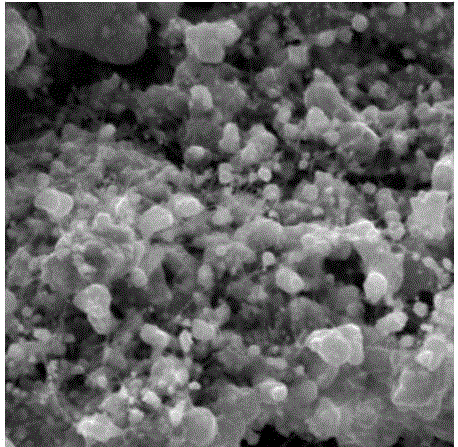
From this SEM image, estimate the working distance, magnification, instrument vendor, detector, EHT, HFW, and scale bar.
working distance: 12.88 mm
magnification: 4.98 K X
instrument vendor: Tescan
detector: SE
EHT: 20 kV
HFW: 41.7 µm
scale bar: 10000 nm